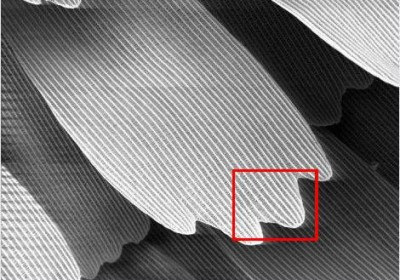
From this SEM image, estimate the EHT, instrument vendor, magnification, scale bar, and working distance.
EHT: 5 kV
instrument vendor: JEOL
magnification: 1 K X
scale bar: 20000 nm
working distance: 19 mm